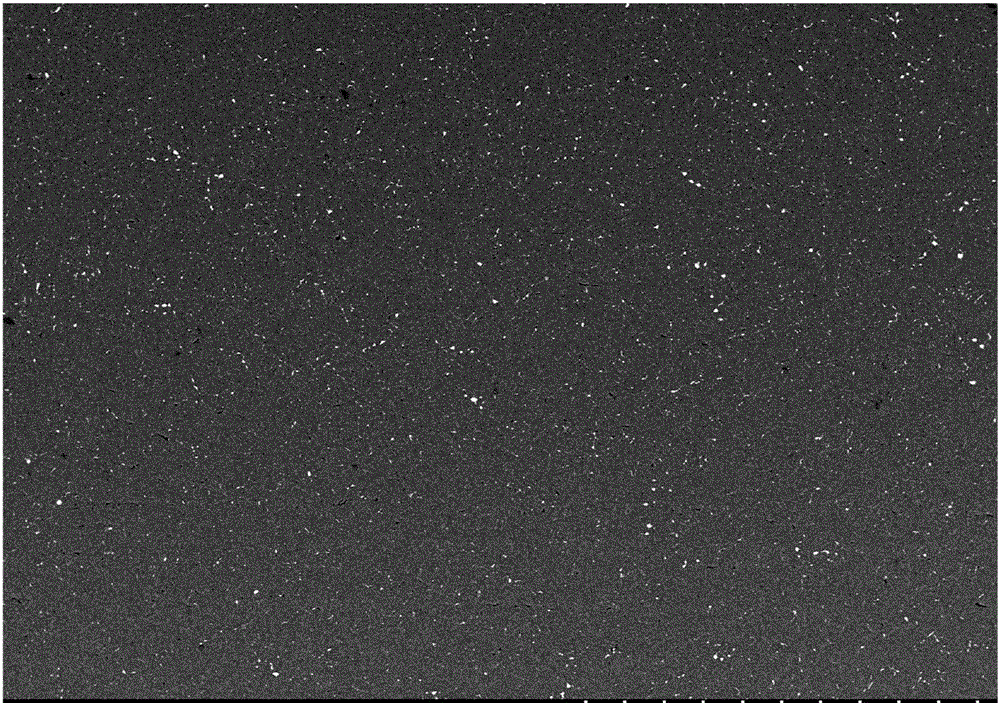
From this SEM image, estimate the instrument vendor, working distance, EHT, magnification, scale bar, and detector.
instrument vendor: Hitachi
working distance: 10.3 mm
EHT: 20 kV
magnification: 0.1 K X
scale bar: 500000 nm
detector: BSE3D